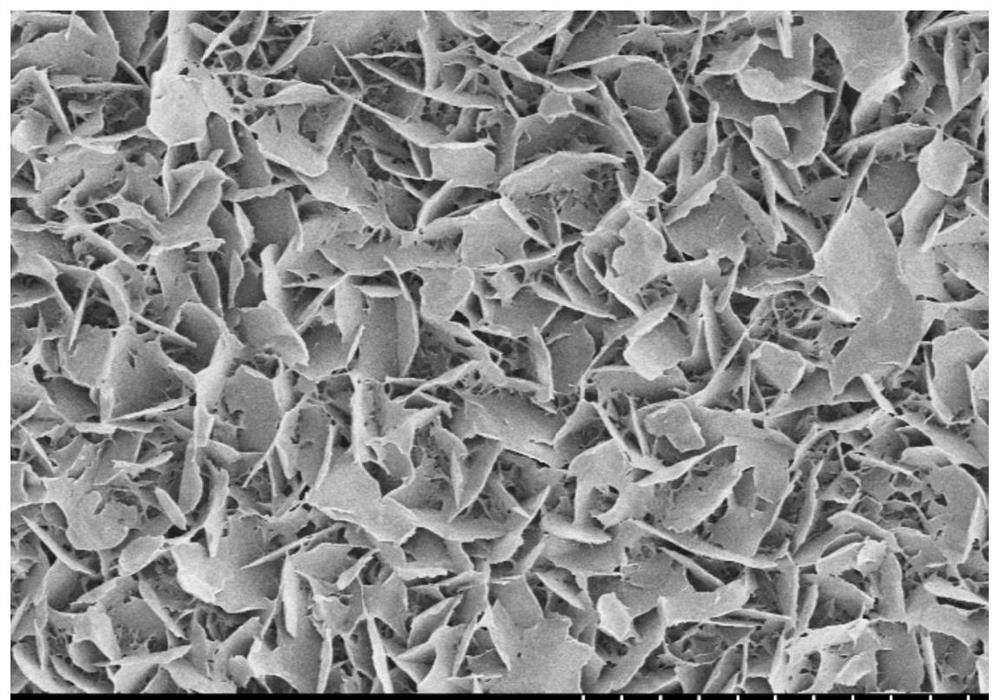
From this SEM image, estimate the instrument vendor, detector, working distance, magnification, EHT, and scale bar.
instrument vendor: Hitachi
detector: SE(M,LA0)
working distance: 10.5 mm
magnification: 1 K X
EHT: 5 kV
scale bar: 50000 nm